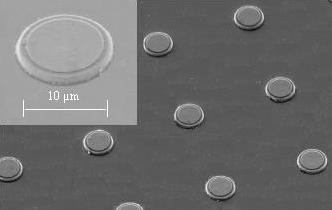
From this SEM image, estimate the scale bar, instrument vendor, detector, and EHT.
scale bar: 10000 nm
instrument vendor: Zeiss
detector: SE1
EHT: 15 kV